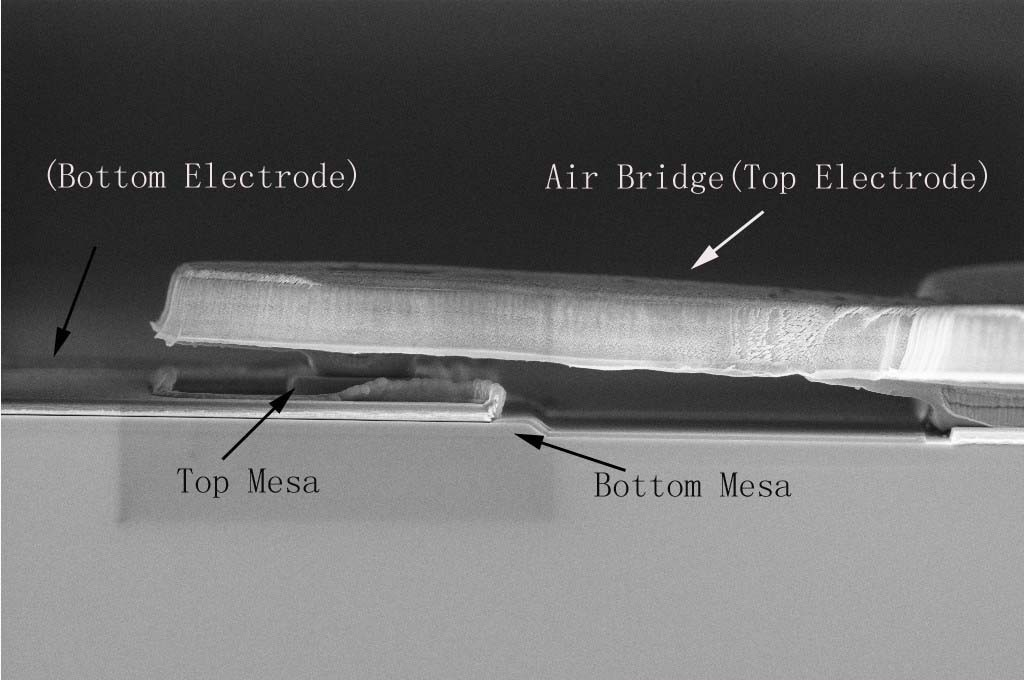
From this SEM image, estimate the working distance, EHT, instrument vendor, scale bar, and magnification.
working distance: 5 mm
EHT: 5 kV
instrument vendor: Zeiss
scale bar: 2000 nm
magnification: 5 K X